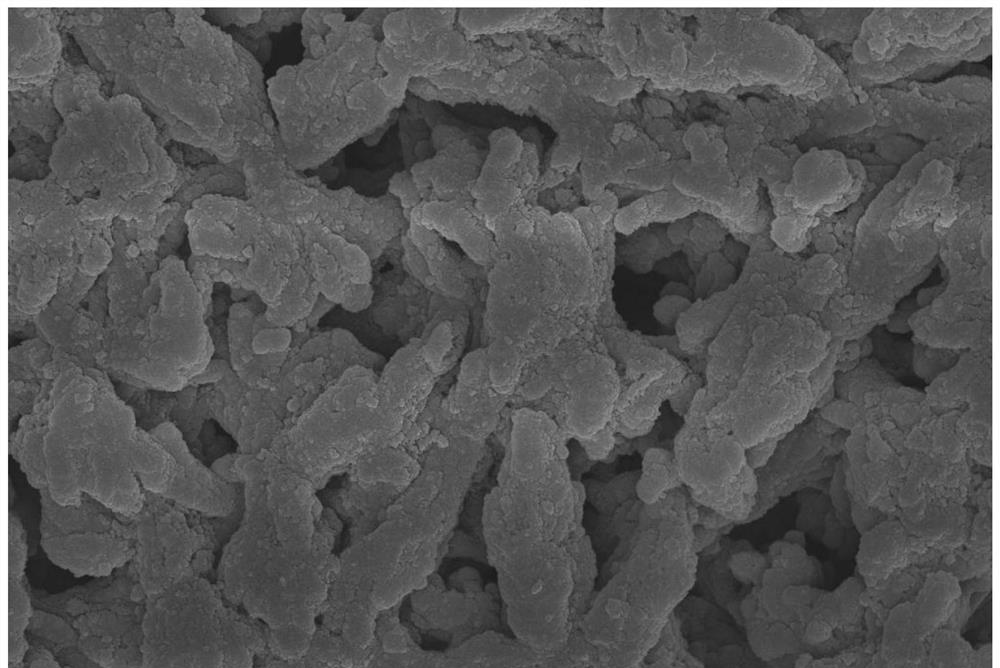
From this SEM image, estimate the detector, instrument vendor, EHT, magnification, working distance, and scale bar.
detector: InLens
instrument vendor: Zeiss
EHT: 10 kV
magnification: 10 K X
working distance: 8.2 mm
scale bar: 1000 nm